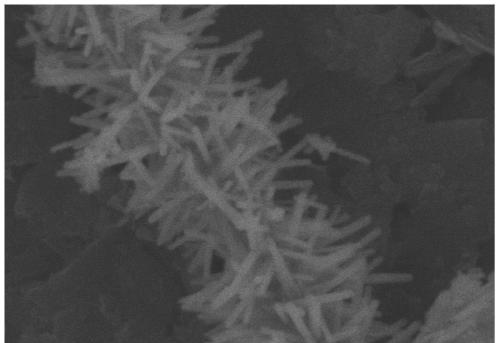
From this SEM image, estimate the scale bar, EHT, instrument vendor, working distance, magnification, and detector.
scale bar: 1000 nm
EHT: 5 kV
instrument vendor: Hitachi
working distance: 10.7 mm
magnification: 50 K X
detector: SE(M)